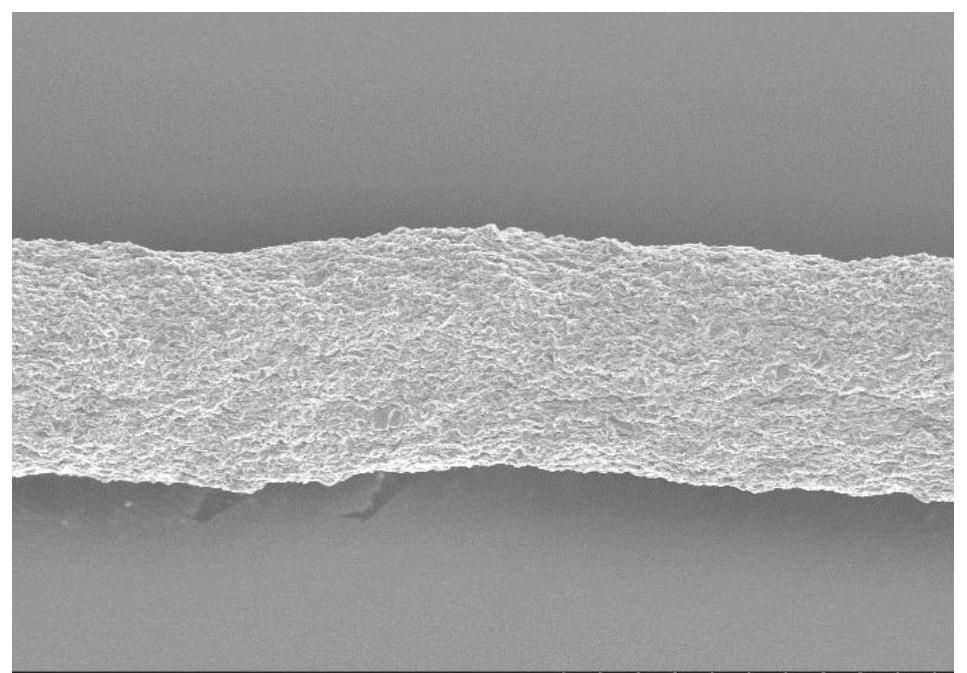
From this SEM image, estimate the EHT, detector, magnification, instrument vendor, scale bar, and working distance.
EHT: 5 kV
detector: SE(M)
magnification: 1 K X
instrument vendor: Hitachi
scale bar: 50000 nm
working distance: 9.9 mm